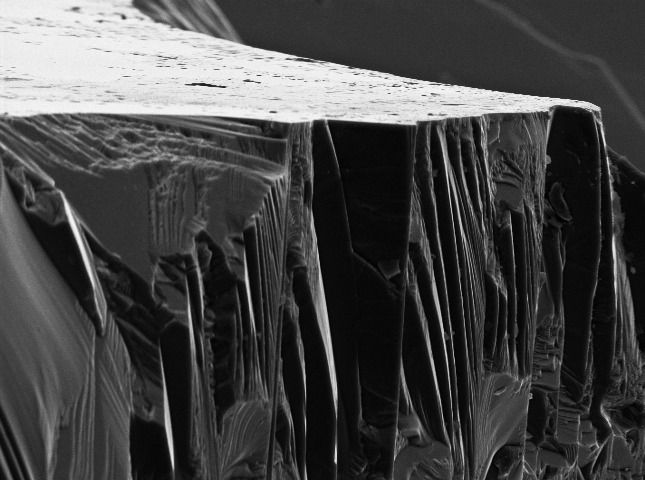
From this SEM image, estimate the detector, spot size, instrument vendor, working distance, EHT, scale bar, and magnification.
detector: SE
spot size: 3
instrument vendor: FEI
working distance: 19.5 mm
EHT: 10 kV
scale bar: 10000 nm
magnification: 2.402 K X